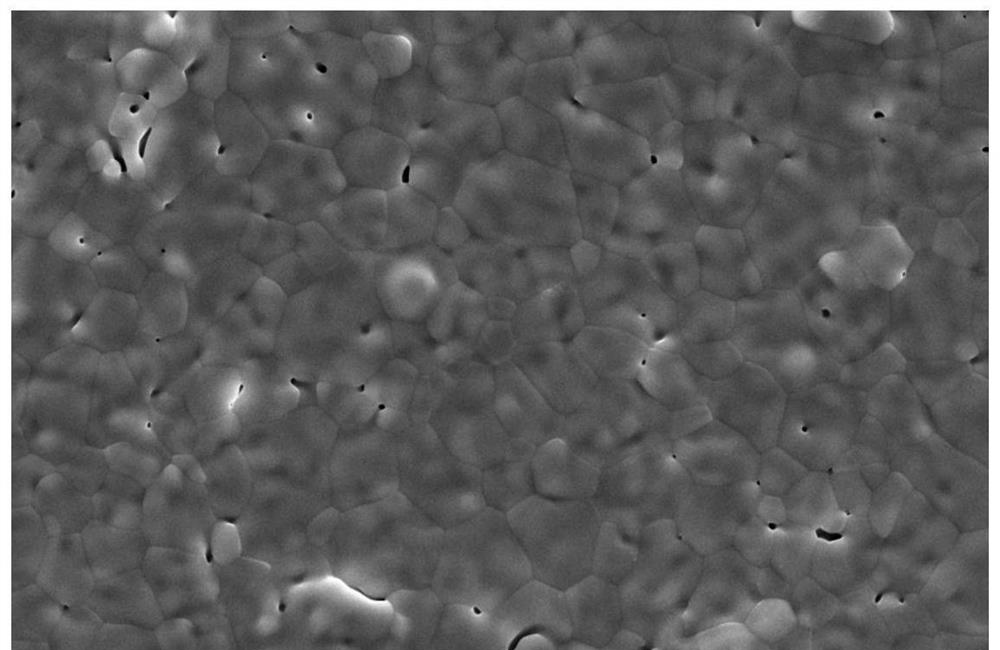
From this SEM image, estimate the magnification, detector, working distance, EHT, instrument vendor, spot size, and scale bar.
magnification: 10 K X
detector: ETD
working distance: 9.6 mm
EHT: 20 kV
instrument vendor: FEI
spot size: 8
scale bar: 10000 nm